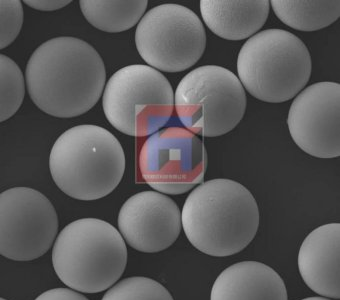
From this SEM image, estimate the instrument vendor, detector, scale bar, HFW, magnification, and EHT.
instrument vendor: other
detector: SED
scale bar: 269000 nm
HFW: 269 µm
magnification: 1 K X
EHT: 10 kV